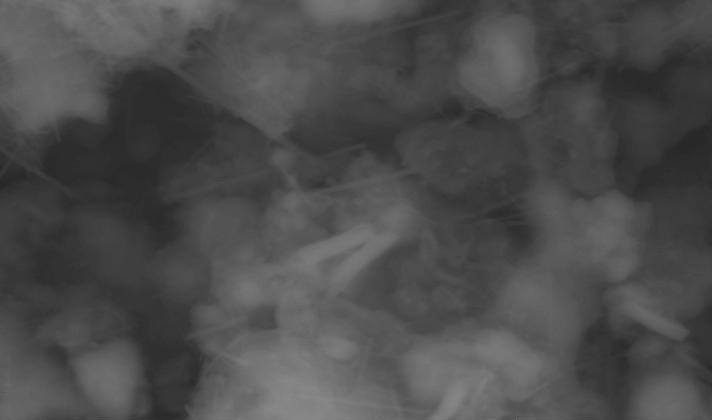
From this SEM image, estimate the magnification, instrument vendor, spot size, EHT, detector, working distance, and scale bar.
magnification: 2.469 K X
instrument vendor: FEI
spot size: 5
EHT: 30 kV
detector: GSE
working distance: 14.1 mm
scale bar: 10000 nm